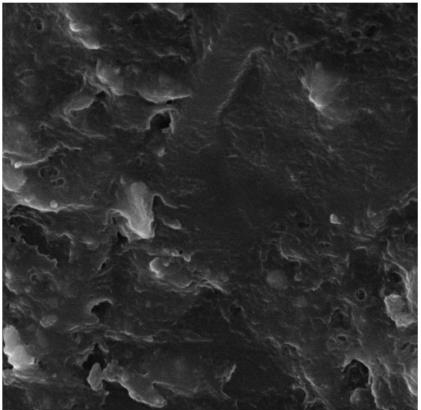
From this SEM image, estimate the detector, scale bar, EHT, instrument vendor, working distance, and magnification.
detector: SE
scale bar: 5000 nm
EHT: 20 kV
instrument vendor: Tescan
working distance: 11.7 mm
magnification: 5.81 K X